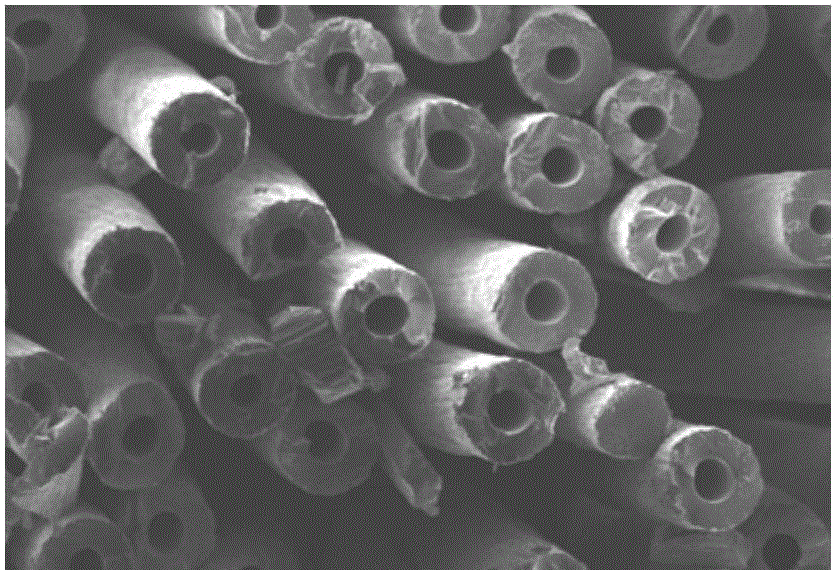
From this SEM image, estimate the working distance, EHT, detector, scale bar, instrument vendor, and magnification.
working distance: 9.6 mm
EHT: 3 kV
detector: SEI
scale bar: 10000 nm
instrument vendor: JEOL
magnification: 1 K X